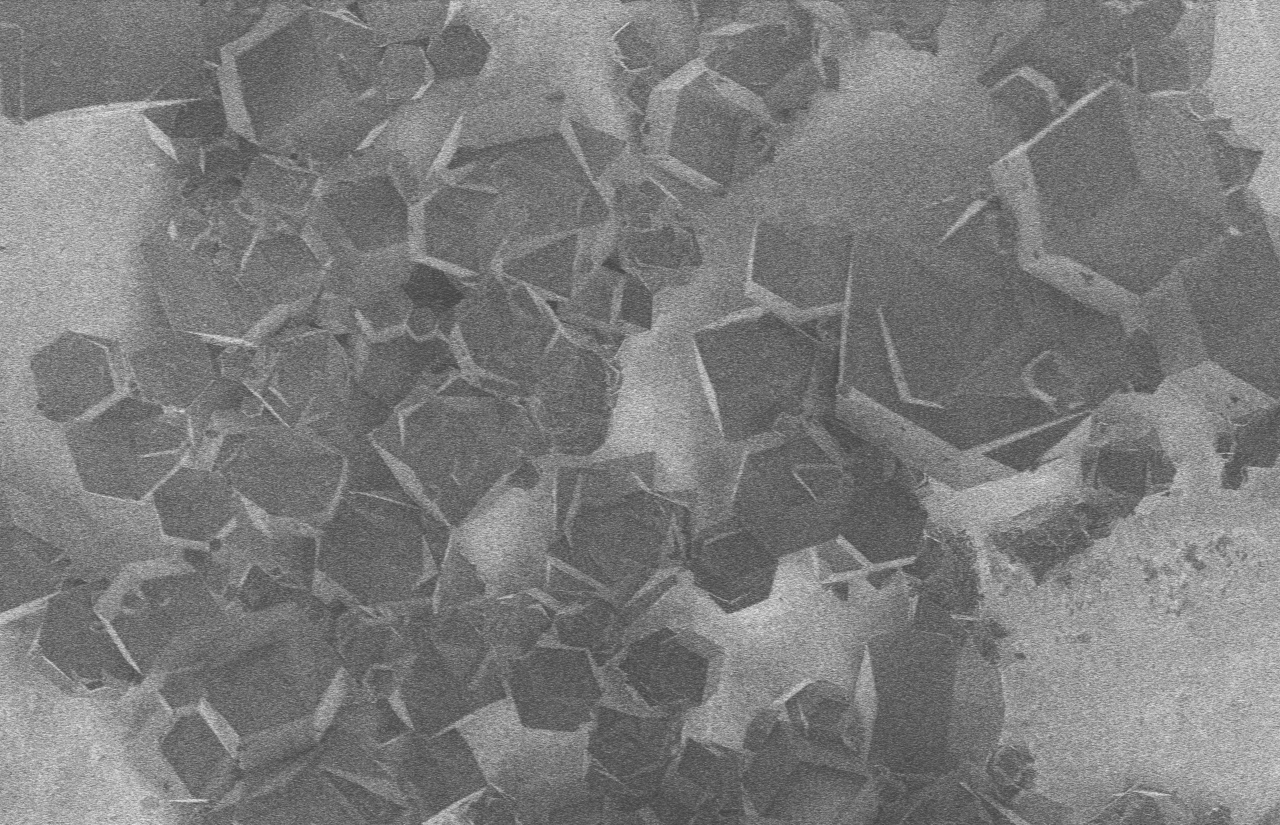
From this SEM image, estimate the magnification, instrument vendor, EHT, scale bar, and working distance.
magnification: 2 K X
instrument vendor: Hitachi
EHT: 1 kV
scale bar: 20000 nm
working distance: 6.1 mm